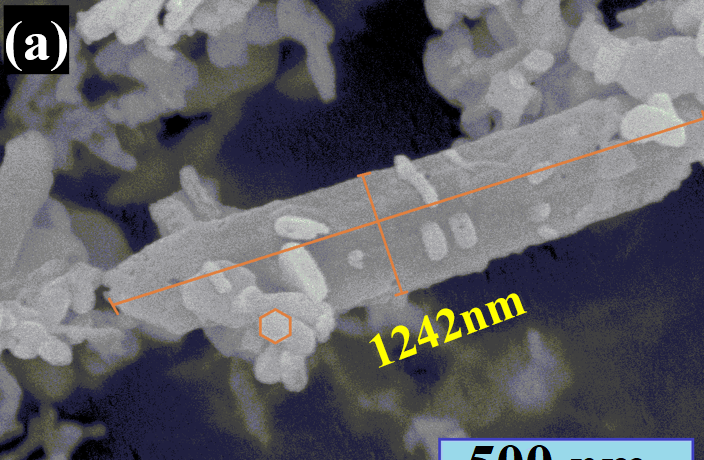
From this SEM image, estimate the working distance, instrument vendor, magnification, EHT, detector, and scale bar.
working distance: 8.2 mm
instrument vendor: Hitachi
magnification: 90 K X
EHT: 5 kV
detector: SE(UL)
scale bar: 500 nm